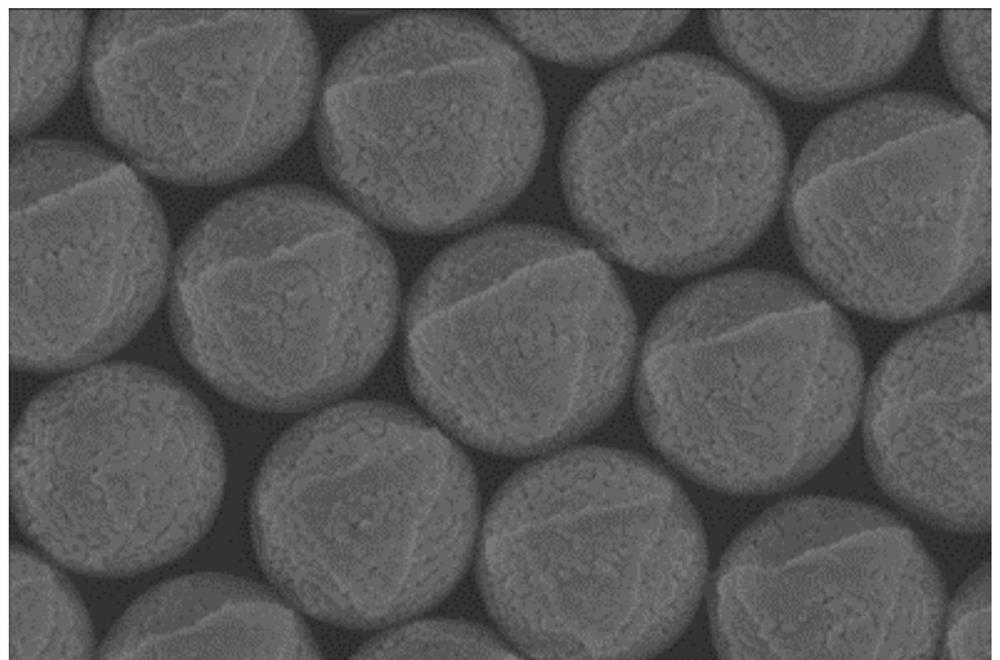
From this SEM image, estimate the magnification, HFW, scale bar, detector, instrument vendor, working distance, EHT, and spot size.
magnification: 51 K X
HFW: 4.06 µm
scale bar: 1000 nm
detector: TLD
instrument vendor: FEI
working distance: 5.3 mm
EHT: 5 kV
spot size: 3